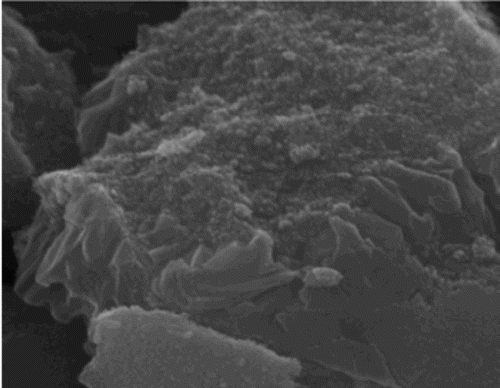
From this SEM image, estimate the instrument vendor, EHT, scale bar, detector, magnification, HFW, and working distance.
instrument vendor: Tescan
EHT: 20 kV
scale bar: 500 nm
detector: SE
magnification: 75 K X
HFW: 2.77 µm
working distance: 9.33 mm